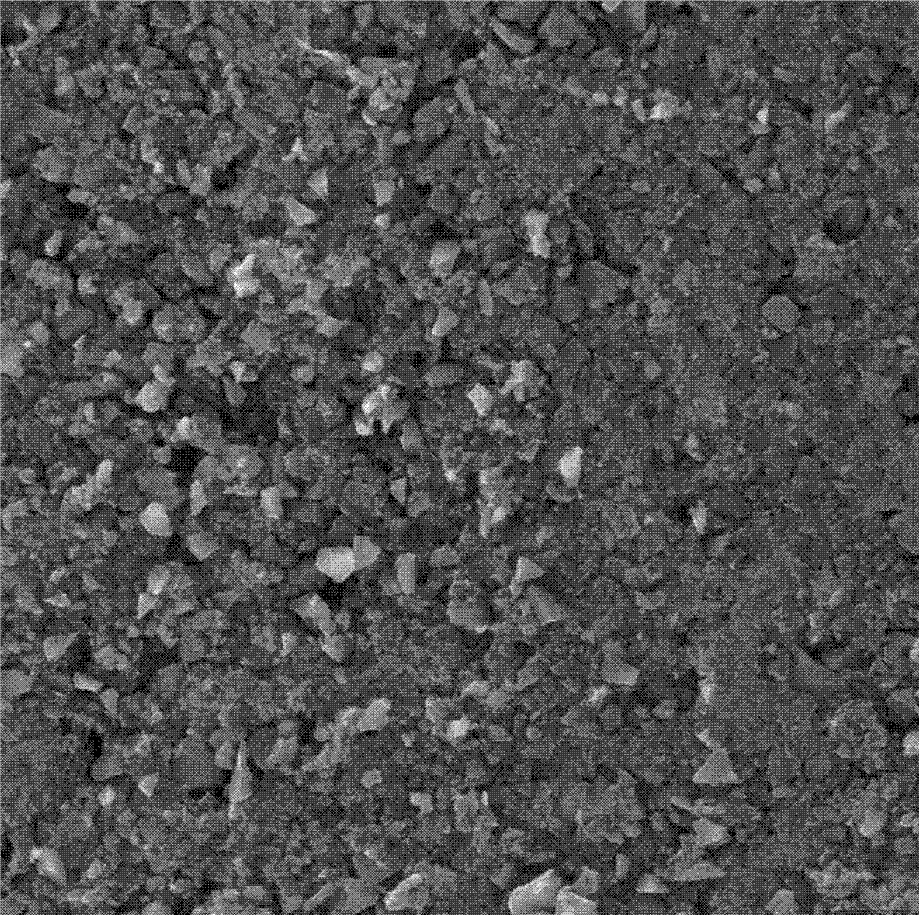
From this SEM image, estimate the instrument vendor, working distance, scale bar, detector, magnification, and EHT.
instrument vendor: Tescan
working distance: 6.8806 mm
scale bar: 100000 nm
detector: SE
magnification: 1 K X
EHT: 30 kV